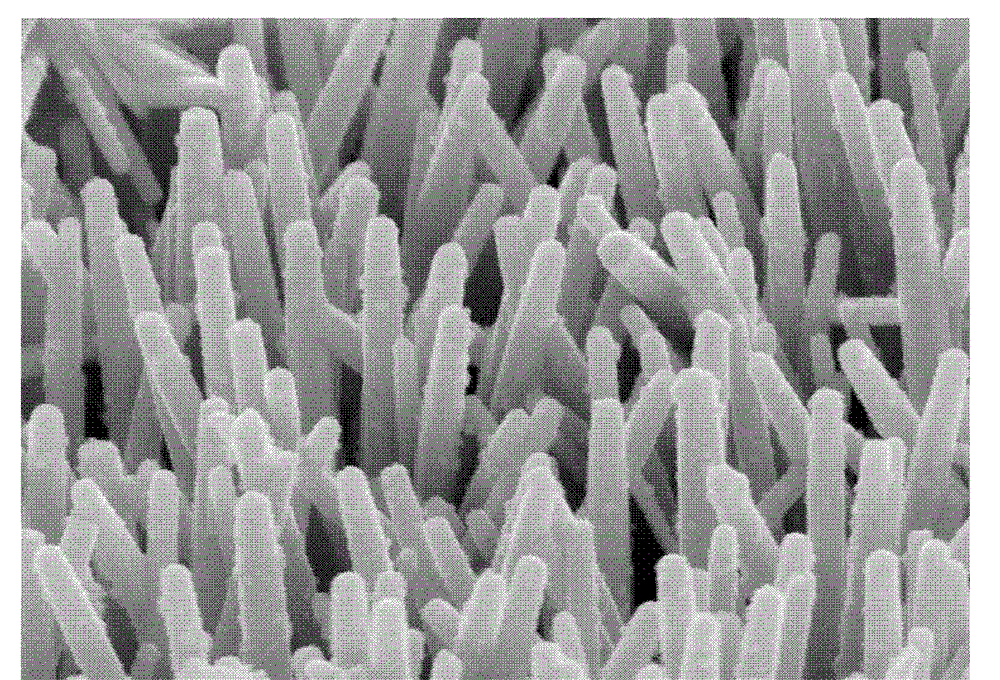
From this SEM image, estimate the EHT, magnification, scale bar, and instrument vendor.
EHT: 5 kV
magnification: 50 K X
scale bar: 1000 nm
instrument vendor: Hitachi